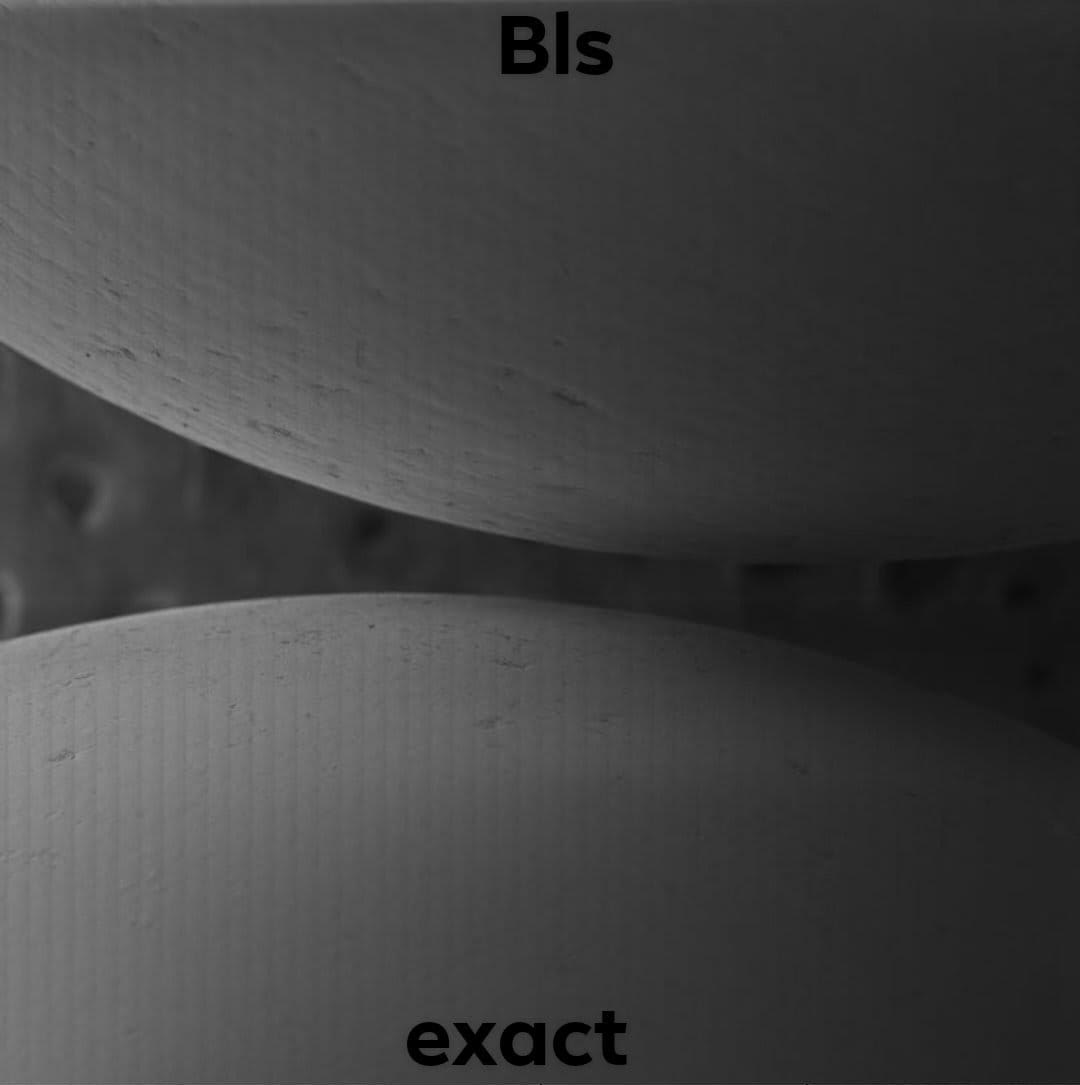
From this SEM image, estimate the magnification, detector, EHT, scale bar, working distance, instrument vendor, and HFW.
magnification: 0.141 K X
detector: SE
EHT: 2 kV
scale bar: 500000 nm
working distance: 14.76 mm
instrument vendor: Tescan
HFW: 2040 µm